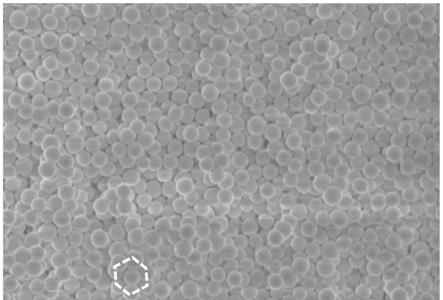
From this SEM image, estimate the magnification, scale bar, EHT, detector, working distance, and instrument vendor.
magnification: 20 K X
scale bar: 2000 nm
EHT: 1 kV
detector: SE(UL)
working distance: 8.1 mm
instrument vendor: Hitachi